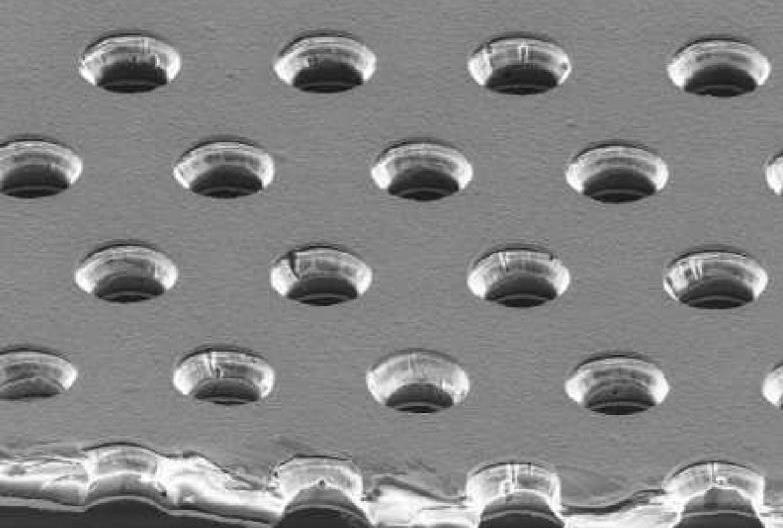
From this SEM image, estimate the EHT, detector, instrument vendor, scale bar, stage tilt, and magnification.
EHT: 15 kV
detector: SE1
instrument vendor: Zeiss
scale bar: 30000 nm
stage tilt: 52°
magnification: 0.2 K X